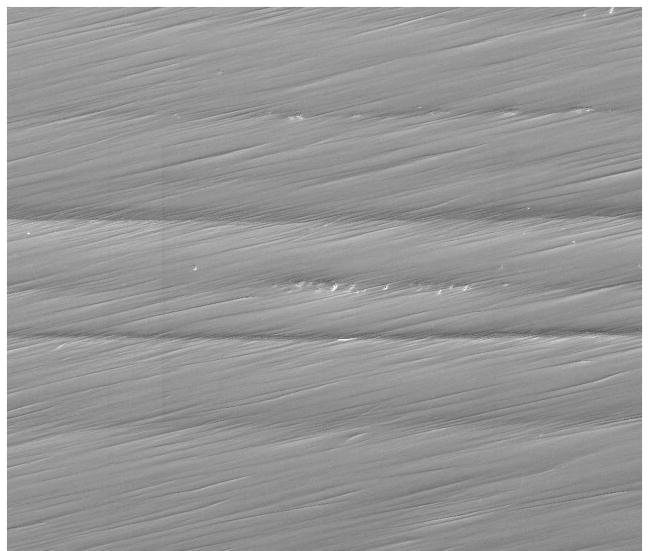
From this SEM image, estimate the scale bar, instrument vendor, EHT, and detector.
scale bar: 400000 nm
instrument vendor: FEI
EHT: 20 kV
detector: ETD